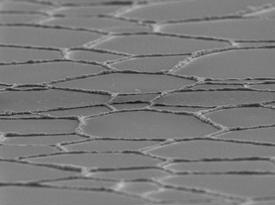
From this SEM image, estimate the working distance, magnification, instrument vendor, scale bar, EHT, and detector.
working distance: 11.8 mm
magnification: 0.5 K X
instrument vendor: JEOL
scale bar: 10000 nm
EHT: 15 kV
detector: SEI